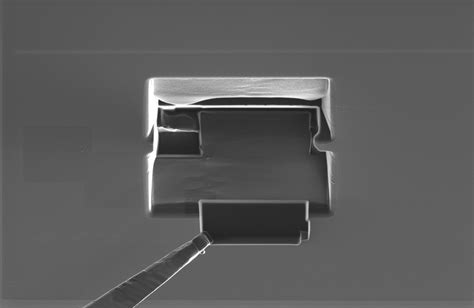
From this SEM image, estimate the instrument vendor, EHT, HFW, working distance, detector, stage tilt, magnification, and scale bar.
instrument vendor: FEI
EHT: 30 kV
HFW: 50.8 µm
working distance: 11 mm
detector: ETD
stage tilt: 0°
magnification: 2.5 K X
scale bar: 10000 nm